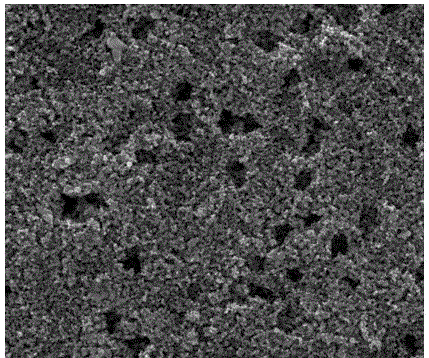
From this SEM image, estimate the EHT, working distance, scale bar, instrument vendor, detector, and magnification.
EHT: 10 kV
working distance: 9.9 mm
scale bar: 2000 nm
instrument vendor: FEI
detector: ETD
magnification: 60 K X